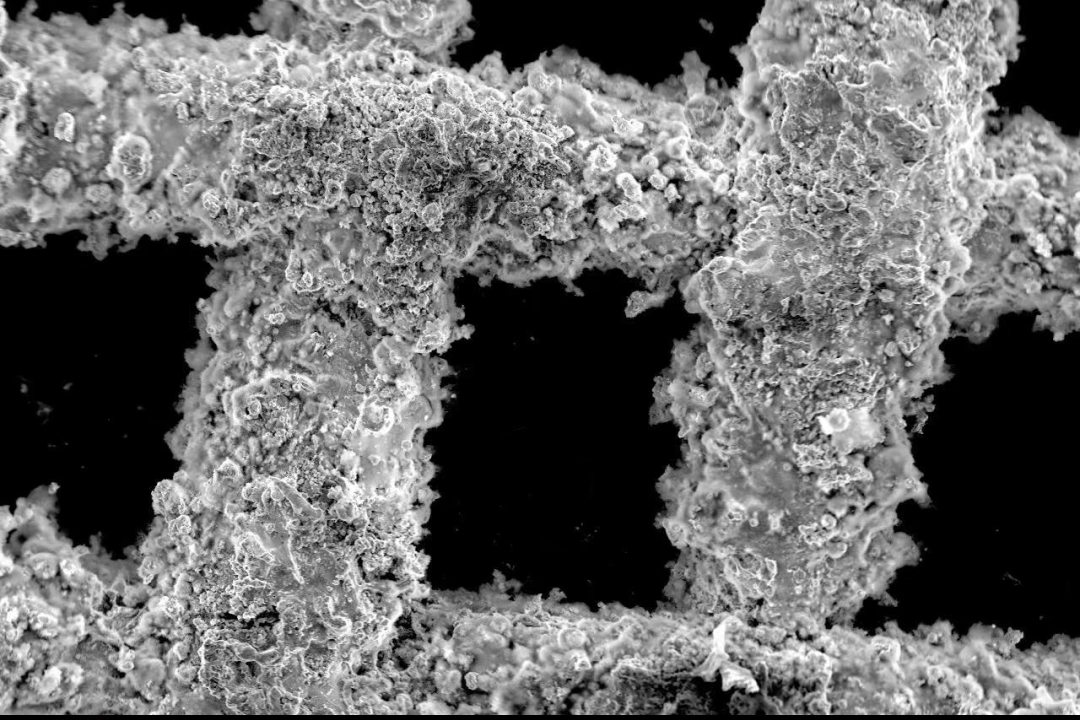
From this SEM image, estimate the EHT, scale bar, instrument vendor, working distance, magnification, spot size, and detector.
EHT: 15 kV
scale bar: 100000 nm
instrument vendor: other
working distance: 13.6 mm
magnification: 0.15 K X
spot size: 14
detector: SE